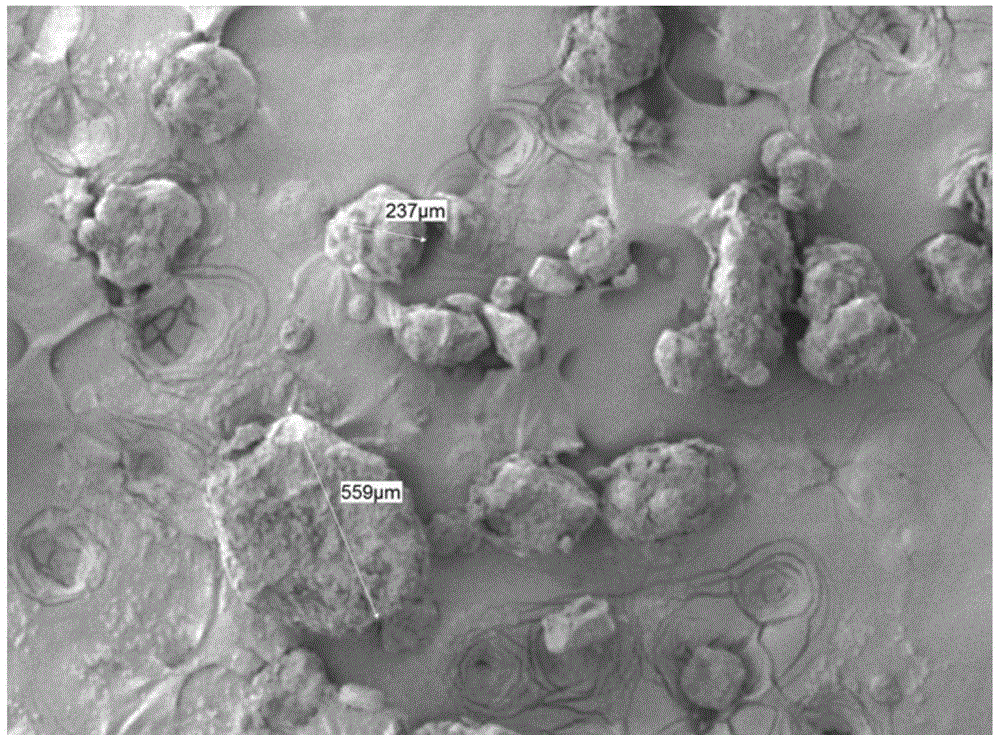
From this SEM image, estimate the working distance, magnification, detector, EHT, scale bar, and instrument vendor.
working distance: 8 mm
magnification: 0.05 K X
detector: LEI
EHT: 2 kV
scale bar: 100000 nm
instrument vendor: JEOL